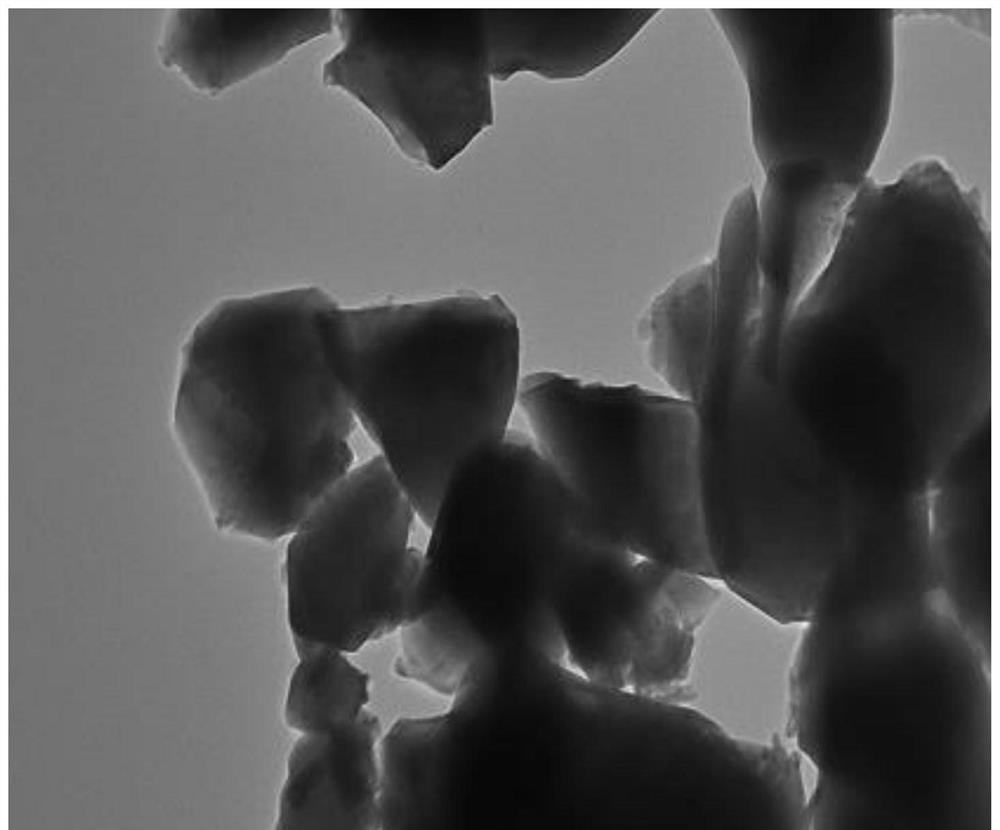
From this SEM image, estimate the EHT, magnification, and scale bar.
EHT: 200 kV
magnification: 40 K X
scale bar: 100 nm